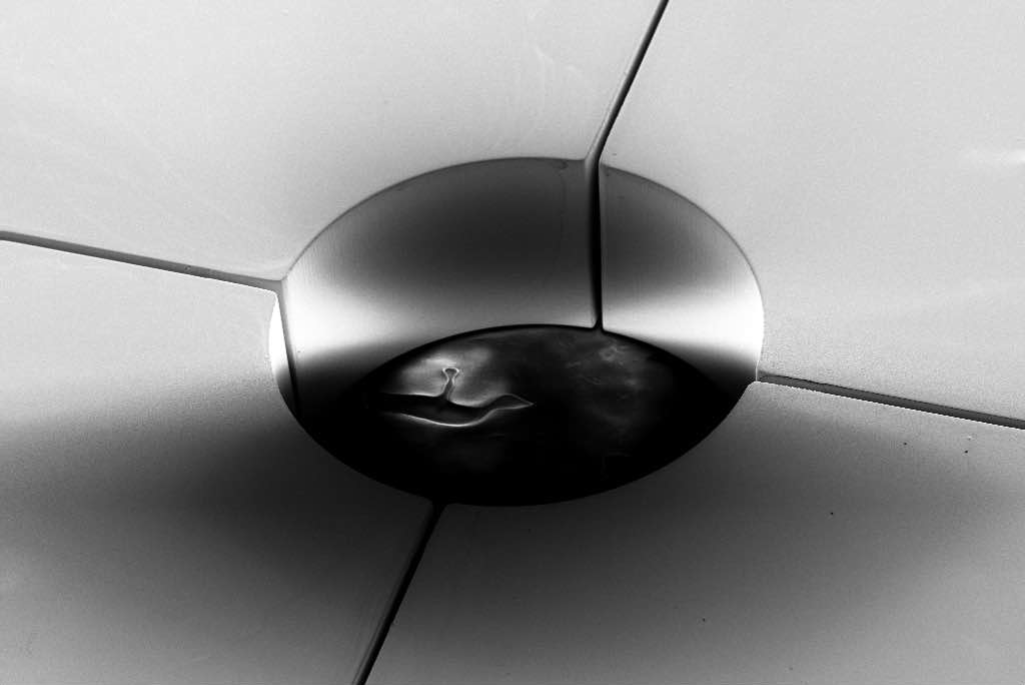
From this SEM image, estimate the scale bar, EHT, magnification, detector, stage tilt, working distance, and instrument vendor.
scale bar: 100000 nm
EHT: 5 kV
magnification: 0.126 K X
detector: SE2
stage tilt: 45°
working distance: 16.6 mm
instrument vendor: Zeiss